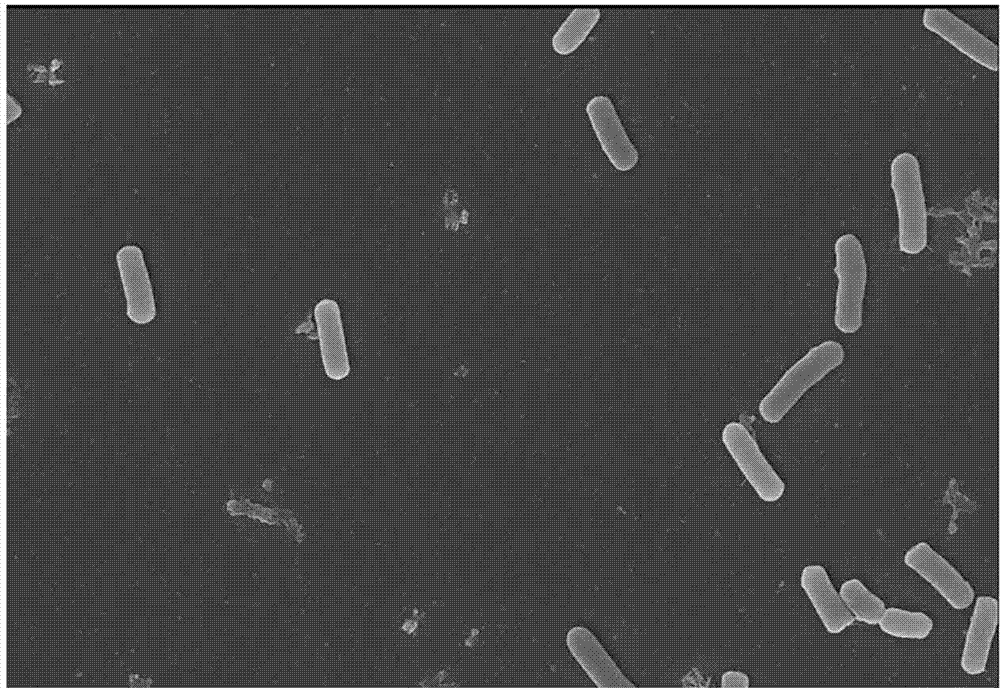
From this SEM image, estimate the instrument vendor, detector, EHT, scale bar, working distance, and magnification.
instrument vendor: Zeiss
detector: InLens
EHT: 10 kV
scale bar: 1000 nm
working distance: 7.5 mm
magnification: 5 K X